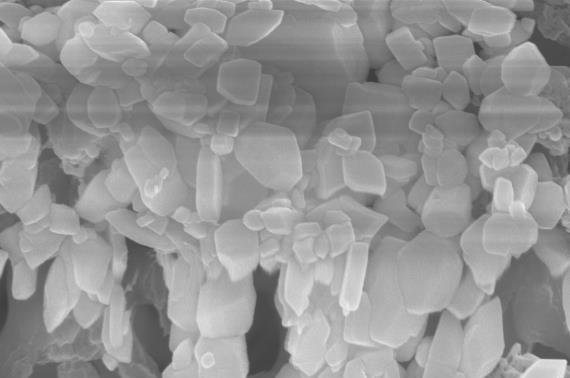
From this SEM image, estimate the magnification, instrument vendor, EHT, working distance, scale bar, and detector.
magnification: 37.63 K X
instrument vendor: Zeiss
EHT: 15 kV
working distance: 4 mm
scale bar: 200 nm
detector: InLens